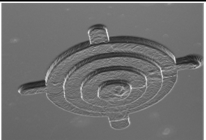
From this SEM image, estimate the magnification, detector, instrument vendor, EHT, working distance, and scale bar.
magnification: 0.05 K X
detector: SE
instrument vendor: Hitachi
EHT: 15 kV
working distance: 31.9 mm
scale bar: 1000000 nm